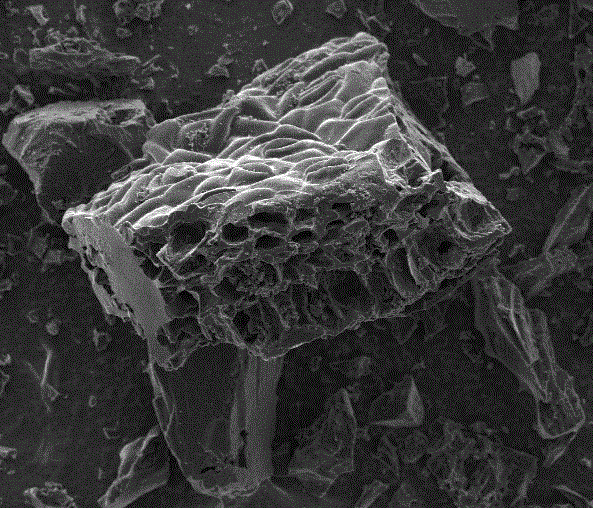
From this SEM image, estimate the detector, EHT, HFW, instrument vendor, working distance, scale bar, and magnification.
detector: ETD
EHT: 5 kV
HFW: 298 µm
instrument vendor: FEI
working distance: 10.2 mm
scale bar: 100000 nm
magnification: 1 K X